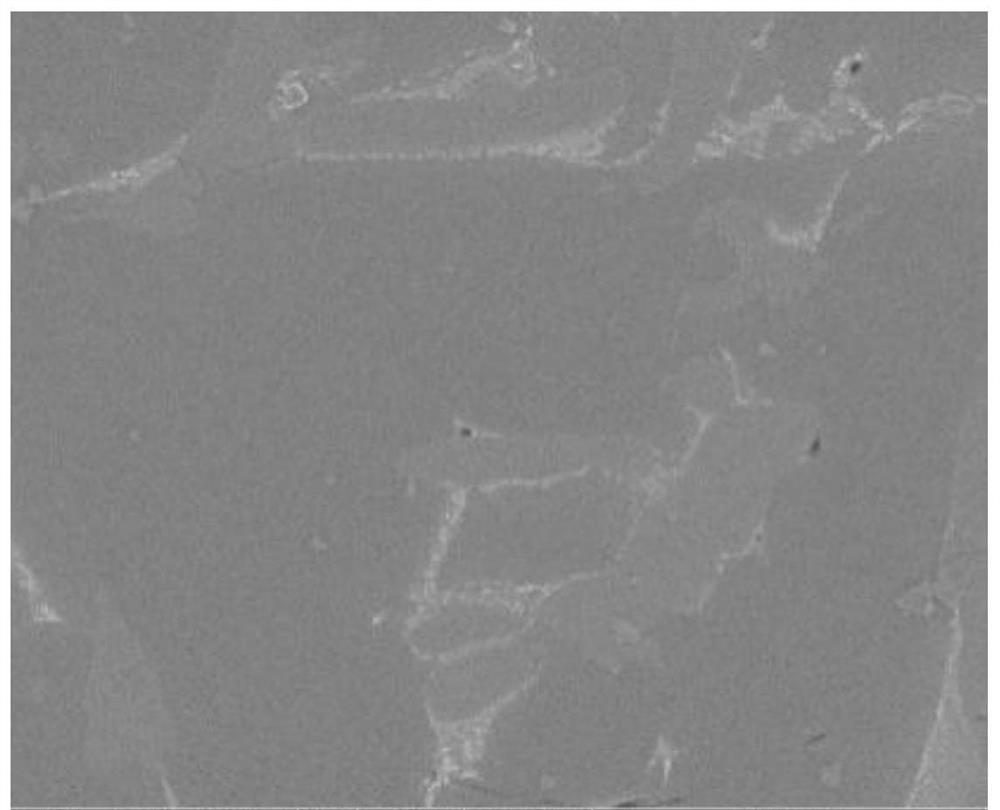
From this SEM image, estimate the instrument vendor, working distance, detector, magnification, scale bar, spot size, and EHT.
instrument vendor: FEI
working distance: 13.3 mm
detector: BSED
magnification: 2 K X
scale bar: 50000 nm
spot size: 4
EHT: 20 kV